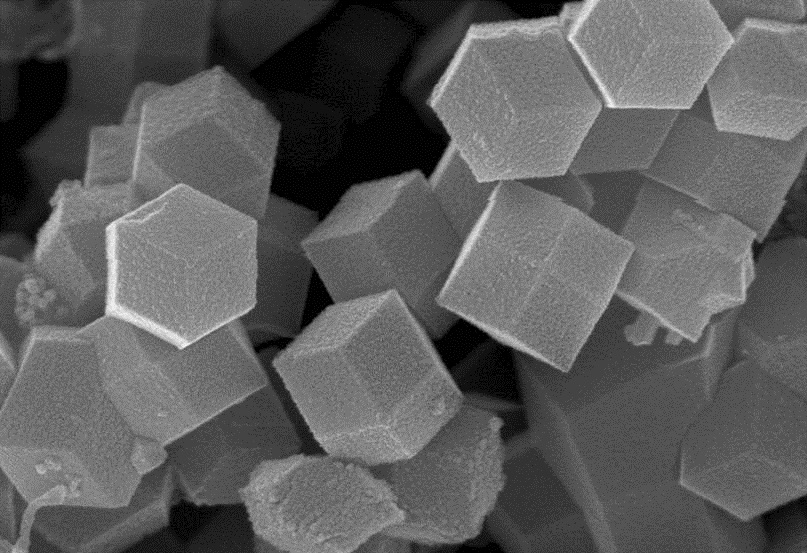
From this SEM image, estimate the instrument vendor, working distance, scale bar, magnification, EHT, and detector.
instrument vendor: Zeiss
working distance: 4.4 mm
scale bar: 200 nm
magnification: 50 K X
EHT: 10 kV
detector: InLens